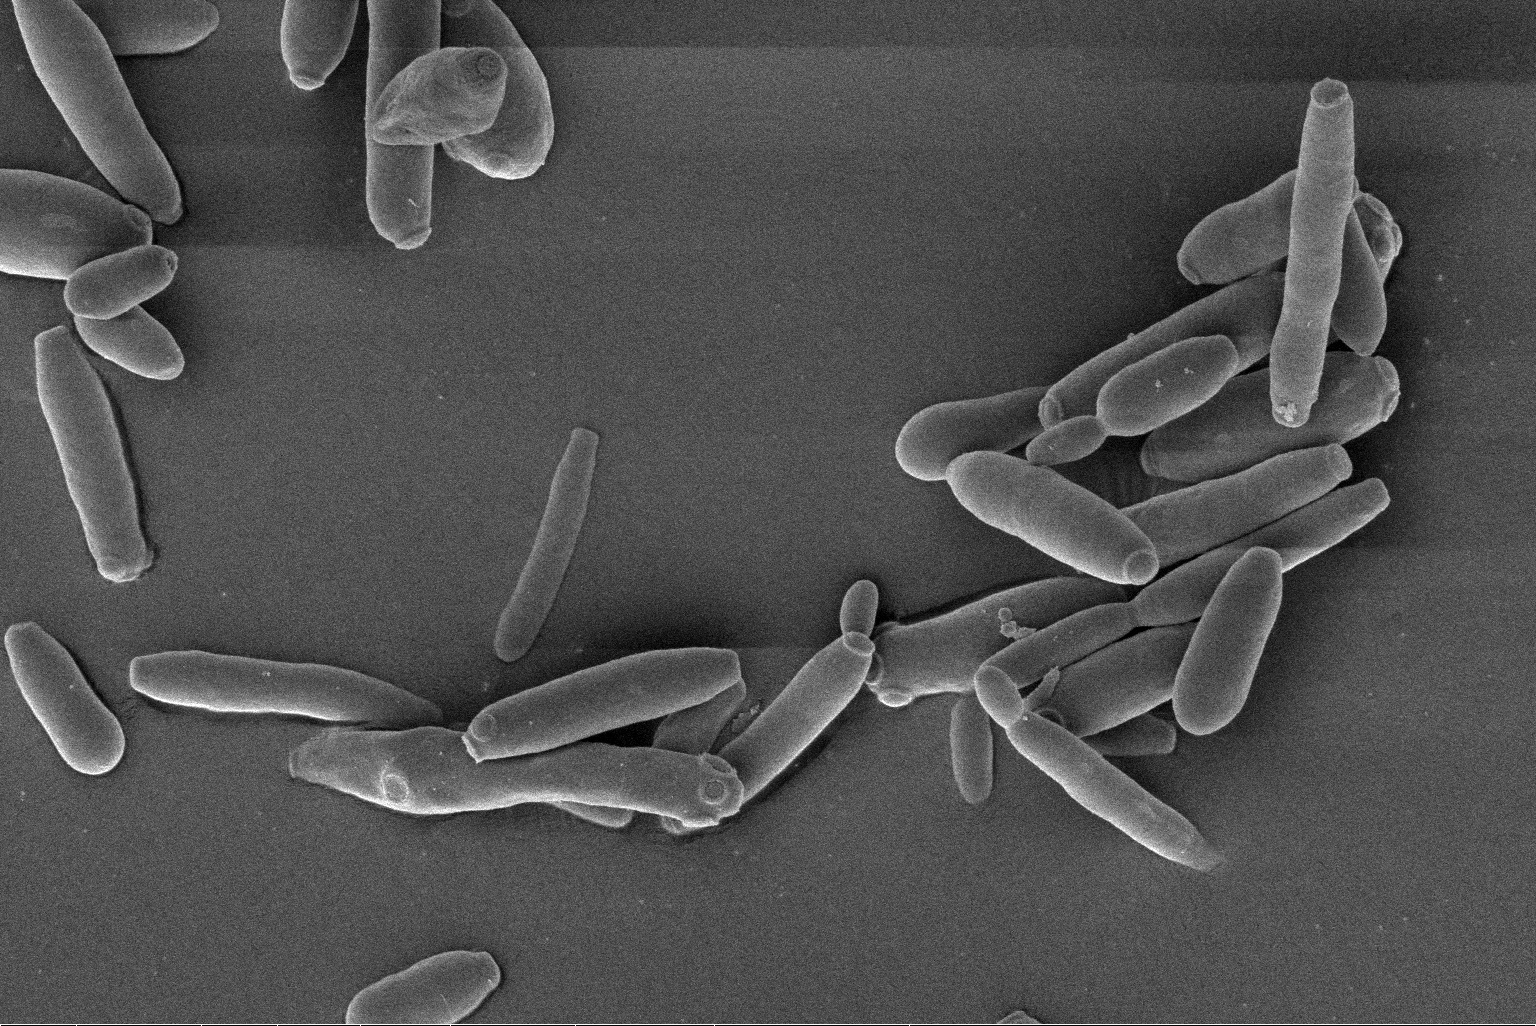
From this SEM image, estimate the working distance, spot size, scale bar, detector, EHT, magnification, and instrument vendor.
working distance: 6.8 mm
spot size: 2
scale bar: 10000 nm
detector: ETD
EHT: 3 kV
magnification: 10 K X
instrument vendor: FEI